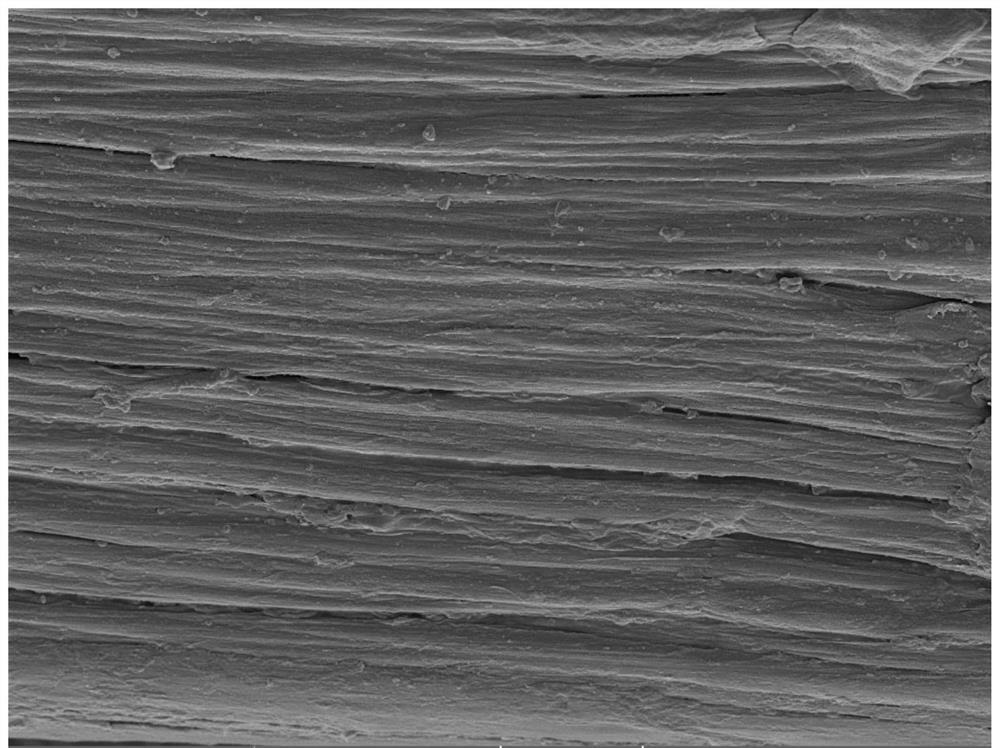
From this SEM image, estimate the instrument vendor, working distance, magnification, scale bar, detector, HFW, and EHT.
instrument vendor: Tescan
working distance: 10.3 mm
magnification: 2 K X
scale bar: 20000 nm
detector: SE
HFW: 138 µm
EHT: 5 kV